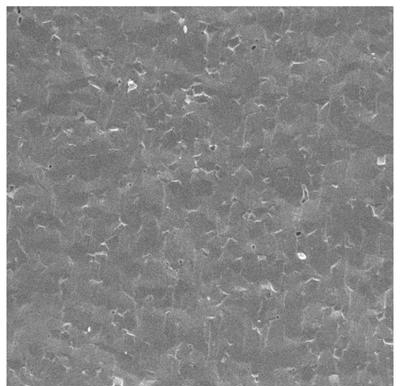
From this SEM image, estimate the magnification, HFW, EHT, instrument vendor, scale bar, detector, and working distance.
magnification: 1 K X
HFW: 284 µm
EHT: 20 kV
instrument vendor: Tescan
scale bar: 50000 nm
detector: SE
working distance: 11.13 mm